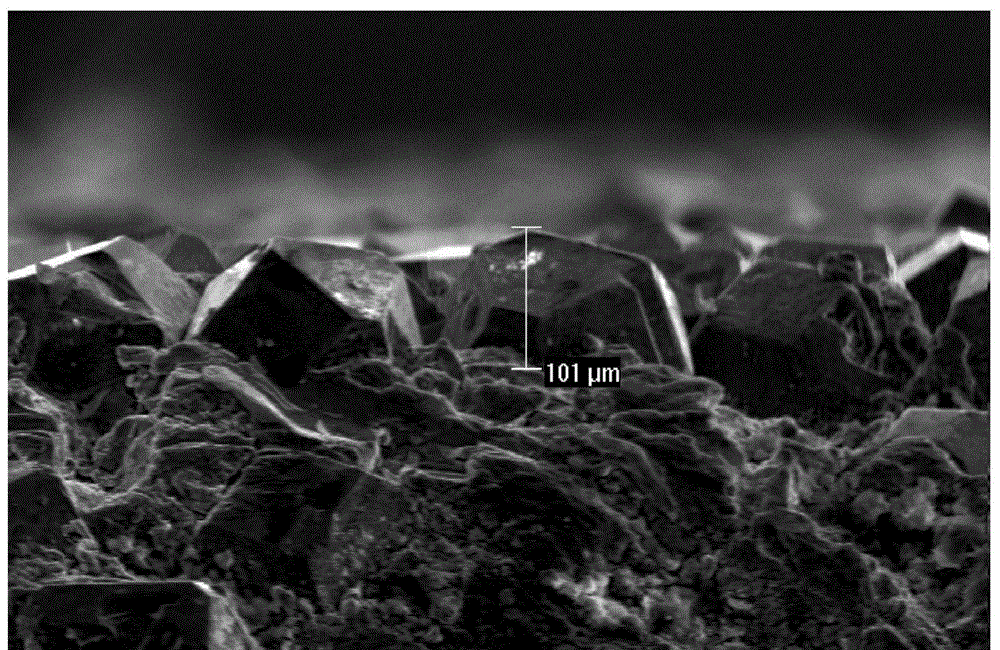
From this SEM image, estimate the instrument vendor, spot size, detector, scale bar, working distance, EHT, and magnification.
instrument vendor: FEI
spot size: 4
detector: SE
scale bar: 200000 nm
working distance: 15.5 mm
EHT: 20 kV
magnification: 0.35 K X